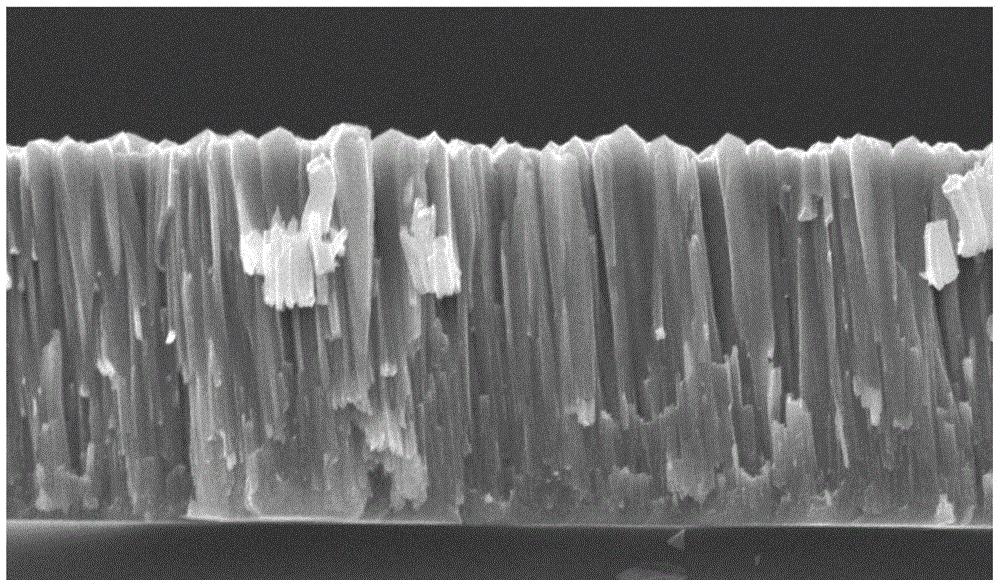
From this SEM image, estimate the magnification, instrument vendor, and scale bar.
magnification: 5 K X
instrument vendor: FEI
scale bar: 5000 nm